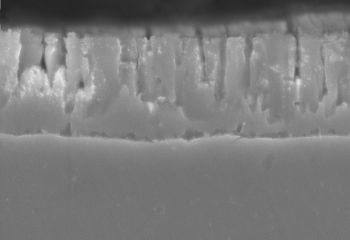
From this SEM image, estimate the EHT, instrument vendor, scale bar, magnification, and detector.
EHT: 25 kV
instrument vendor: Hitachi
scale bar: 10000 nm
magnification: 5 K X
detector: SE